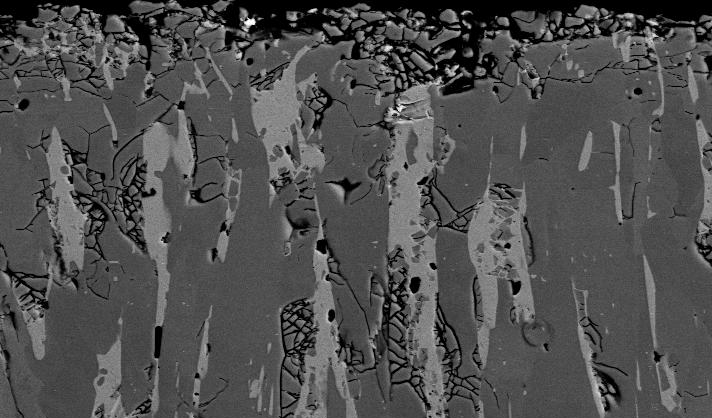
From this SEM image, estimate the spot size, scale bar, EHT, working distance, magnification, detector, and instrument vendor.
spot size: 3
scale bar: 20000 nm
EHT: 20 kV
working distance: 6.3 mm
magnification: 2 K X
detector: BSE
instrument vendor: FEI